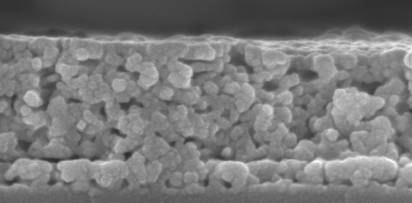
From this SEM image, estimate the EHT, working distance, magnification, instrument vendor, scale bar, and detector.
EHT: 5 kV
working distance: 7.9 mm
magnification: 180 K X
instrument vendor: Hitachi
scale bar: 300 nm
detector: SE(U)